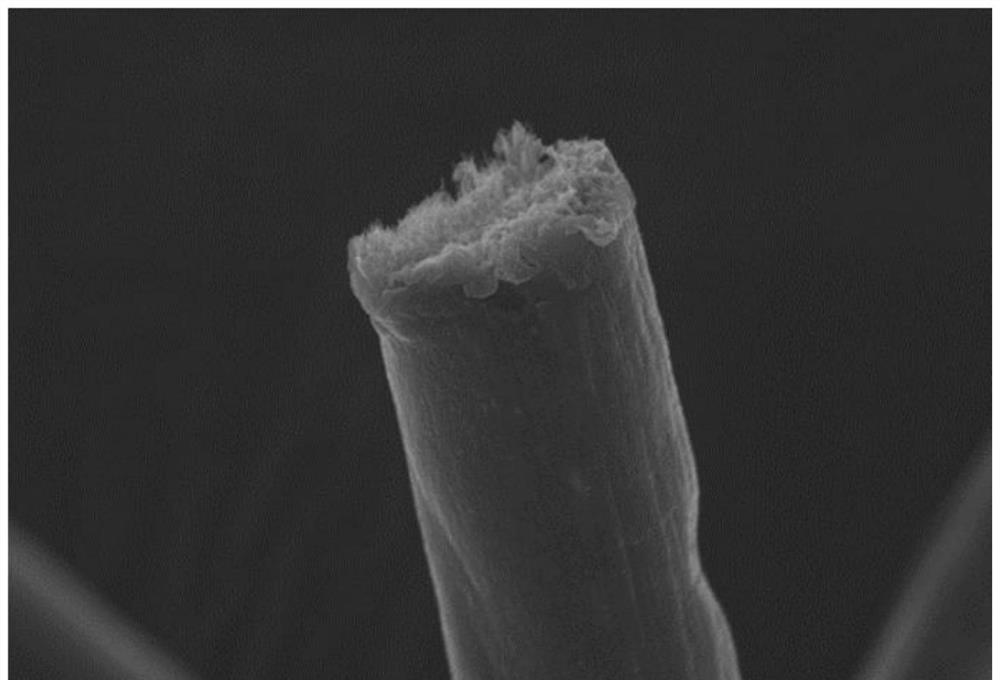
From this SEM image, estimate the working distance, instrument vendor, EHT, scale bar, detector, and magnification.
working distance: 8.9 mm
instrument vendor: Hitachi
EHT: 5 kV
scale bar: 5000 nm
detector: SE(UL)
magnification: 7 K X